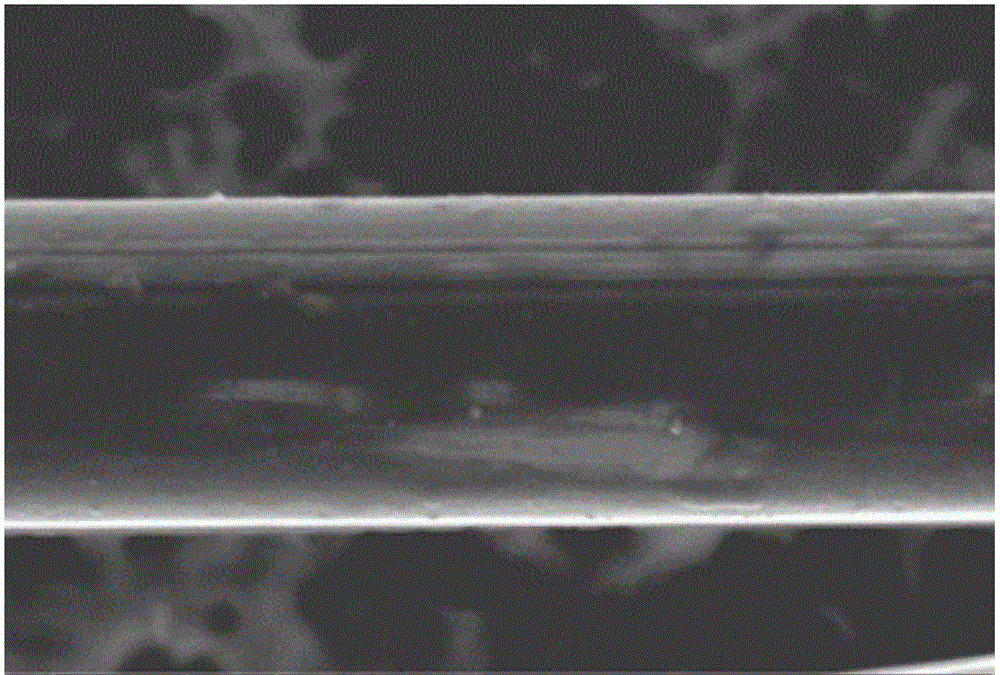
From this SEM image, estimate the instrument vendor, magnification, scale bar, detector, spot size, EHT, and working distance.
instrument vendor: FEI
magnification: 20 K X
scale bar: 10000 nm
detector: TLD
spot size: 3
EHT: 5 kV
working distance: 6.1 mm